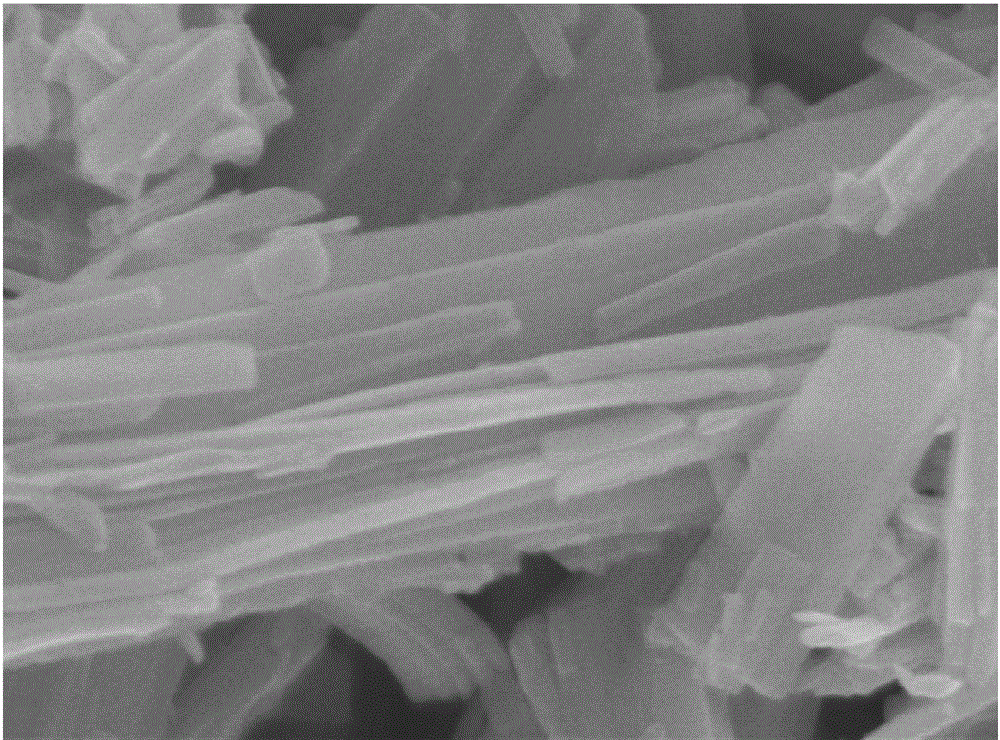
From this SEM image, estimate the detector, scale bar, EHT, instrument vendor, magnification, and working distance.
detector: SE(U)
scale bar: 500 nm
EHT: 5 kV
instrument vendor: Hitachi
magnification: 100 K X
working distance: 8.2 mm